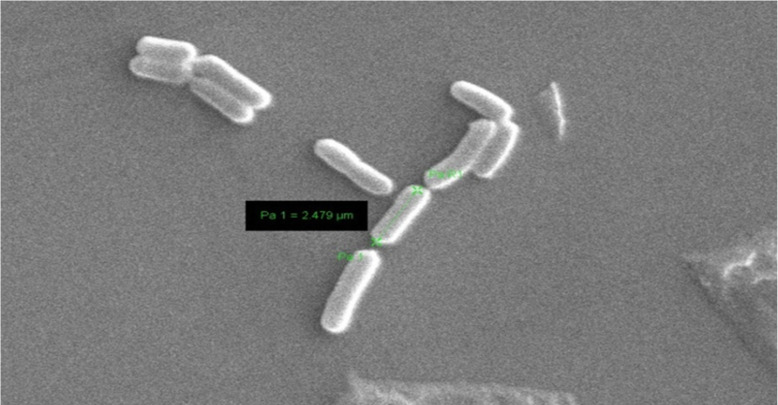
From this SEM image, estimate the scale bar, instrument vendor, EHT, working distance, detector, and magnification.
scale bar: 2000 nm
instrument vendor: Zeiss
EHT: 20 kV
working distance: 8.5 mm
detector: SE1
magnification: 12 K X